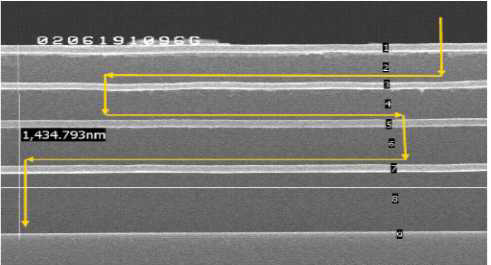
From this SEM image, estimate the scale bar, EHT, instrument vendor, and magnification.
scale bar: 667 nm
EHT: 20 kV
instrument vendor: JEOL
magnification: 45 K X